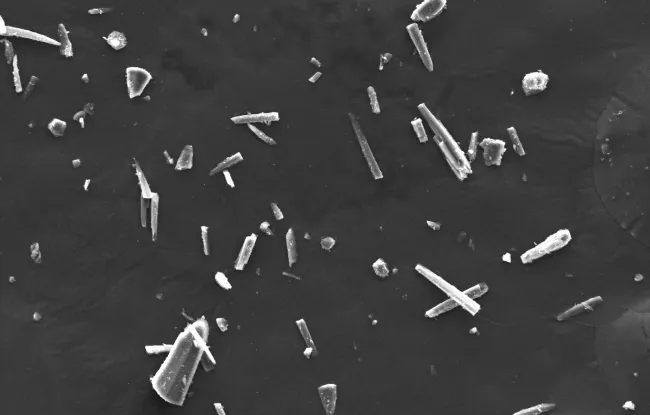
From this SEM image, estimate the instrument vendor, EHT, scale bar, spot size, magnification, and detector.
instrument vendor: Zeiss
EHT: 20 kV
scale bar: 20000 nm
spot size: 325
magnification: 0.617 K X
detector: SE1